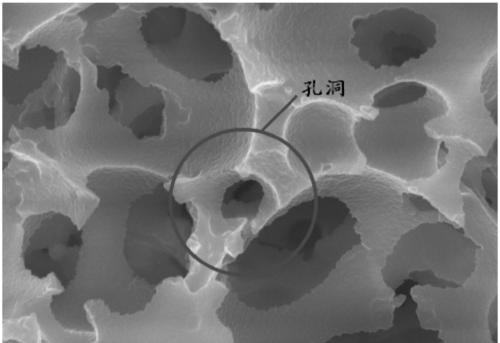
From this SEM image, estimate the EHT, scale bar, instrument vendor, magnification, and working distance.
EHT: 20 kV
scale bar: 5000 nm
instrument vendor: Hitachi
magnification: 8 K X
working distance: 11.8 mm